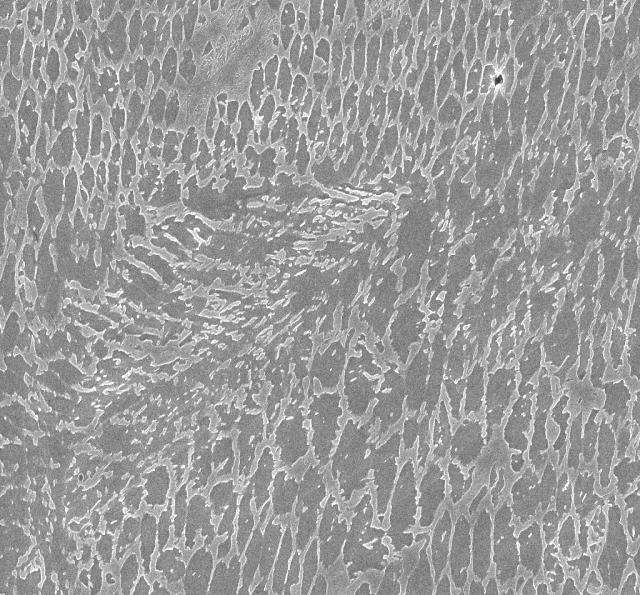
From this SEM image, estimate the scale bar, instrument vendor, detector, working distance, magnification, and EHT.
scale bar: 2000 nm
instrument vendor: Zeiss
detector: InLens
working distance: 8 mm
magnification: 10 K X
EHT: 15 kV